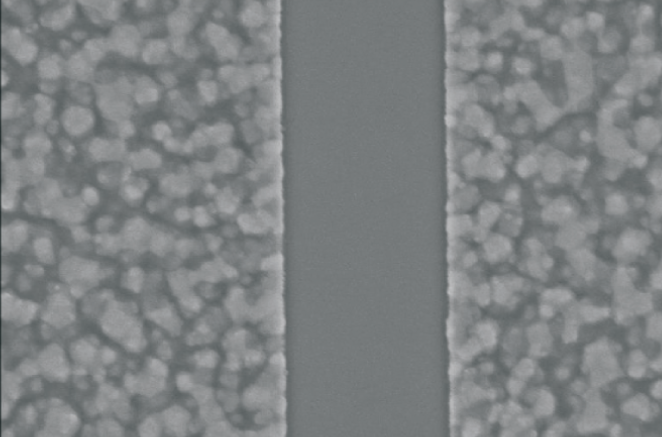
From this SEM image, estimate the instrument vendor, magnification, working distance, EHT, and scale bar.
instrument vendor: FEI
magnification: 35 K X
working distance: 5.5 mm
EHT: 10 kV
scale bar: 5000 nm